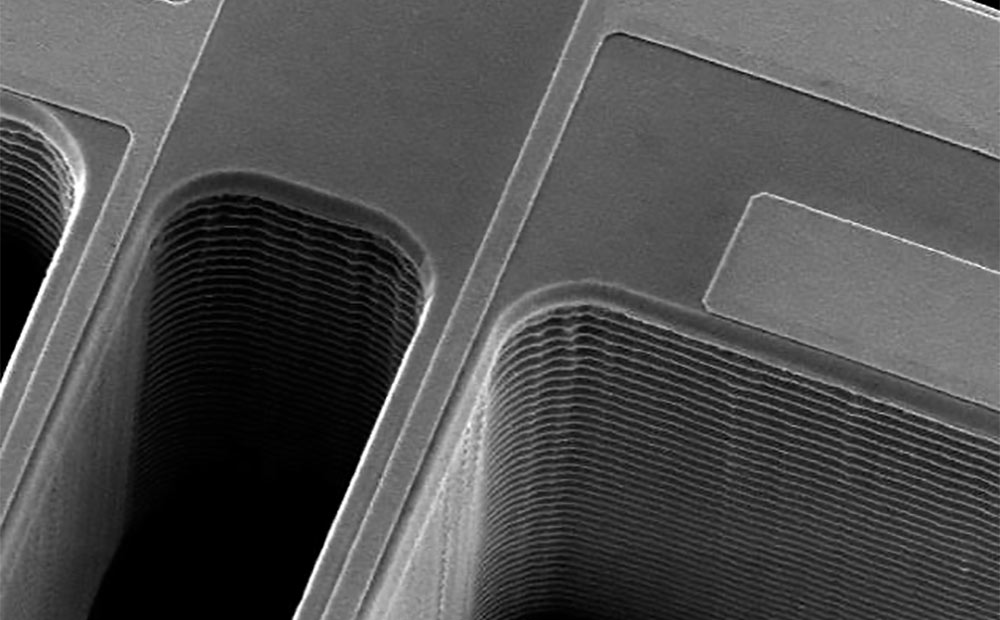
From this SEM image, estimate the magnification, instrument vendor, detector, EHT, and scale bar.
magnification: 1.2 K X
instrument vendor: JEOL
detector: SEI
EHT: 20 kV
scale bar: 10000 nm